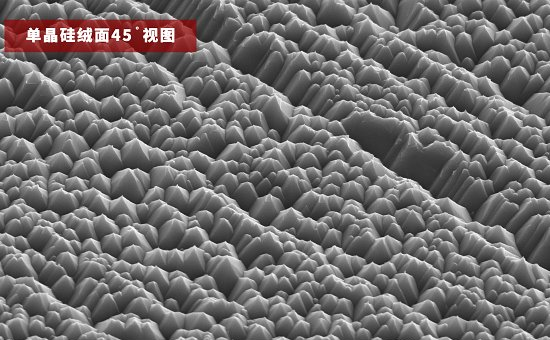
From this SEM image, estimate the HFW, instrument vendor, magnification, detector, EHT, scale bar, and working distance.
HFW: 25.4 µm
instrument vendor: Thermo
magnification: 5 K X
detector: SED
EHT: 10 kV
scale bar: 8000 nm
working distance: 9.434 mm